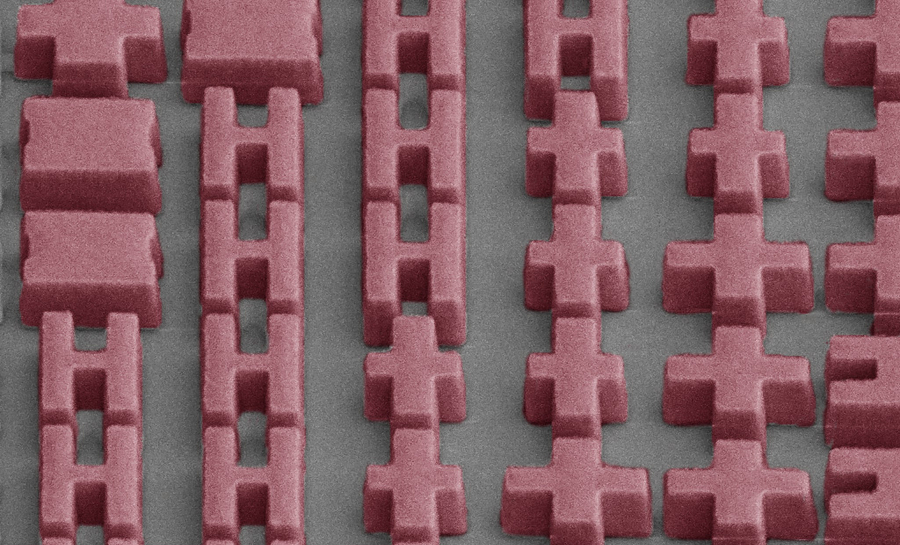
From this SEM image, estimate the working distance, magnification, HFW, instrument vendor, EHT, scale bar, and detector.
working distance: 4 mm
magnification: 8 K X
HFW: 16 µm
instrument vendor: FEI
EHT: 5 kV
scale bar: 5000 nm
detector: ETD